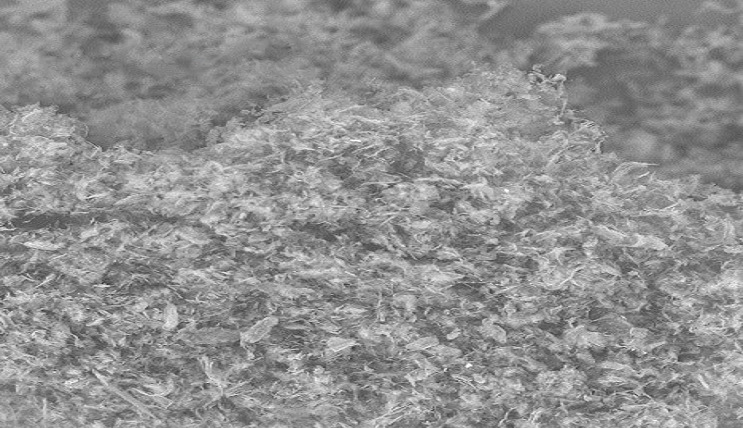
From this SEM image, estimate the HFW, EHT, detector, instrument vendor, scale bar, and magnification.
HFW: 288 µm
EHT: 10 kV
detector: SH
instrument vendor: other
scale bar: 80000 nm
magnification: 0.94 K X